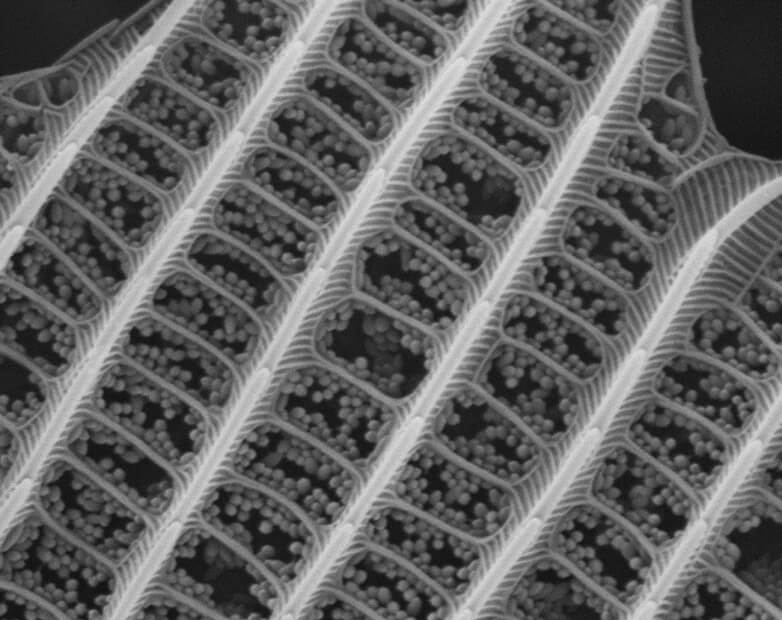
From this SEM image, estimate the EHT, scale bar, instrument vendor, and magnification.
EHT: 15 kV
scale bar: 2000 nm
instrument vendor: JEOL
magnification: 10 K X